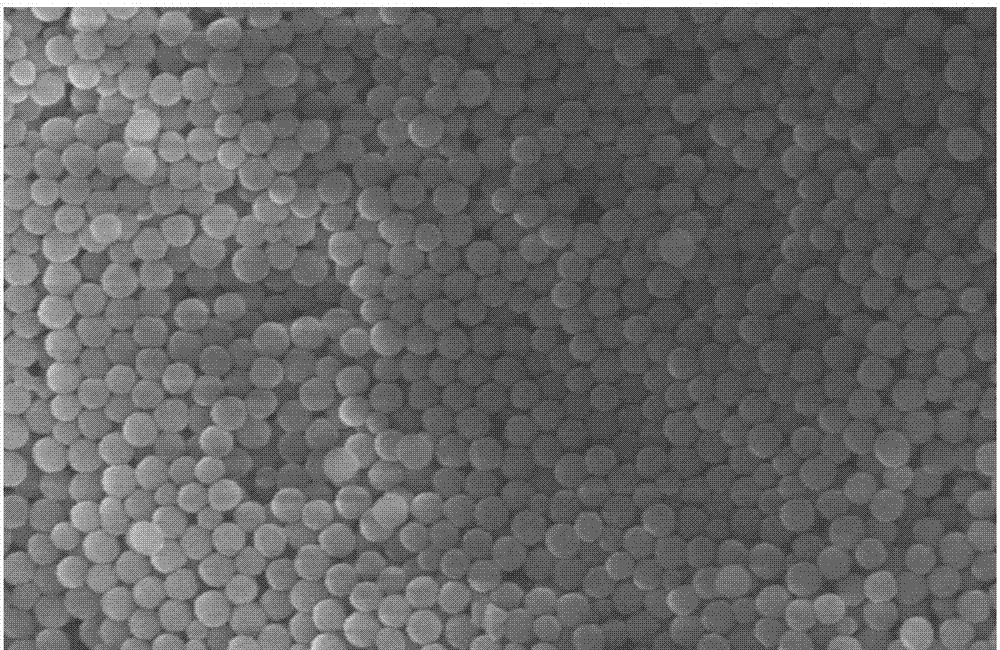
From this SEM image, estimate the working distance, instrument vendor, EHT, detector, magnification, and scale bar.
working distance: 8.5 mm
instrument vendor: Hitachi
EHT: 5 kV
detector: SE(M)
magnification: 25 K X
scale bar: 2000 nm